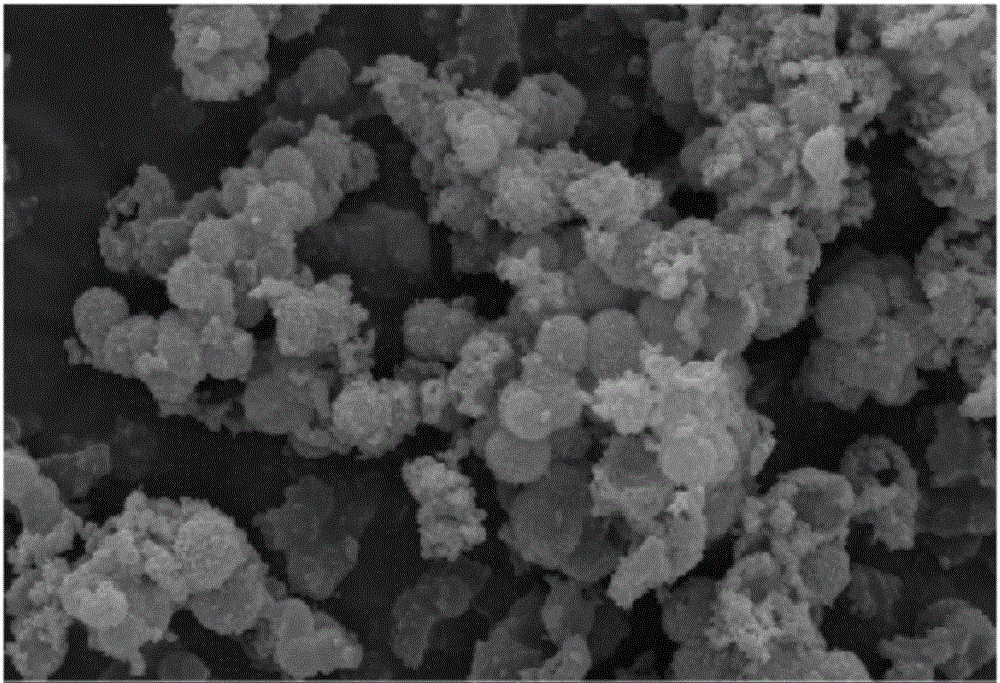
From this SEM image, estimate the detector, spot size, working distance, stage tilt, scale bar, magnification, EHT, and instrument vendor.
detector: ETD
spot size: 4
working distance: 9.1 mm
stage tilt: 0°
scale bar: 5000 nm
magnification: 25 K X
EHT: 20 kV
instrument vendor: FEI